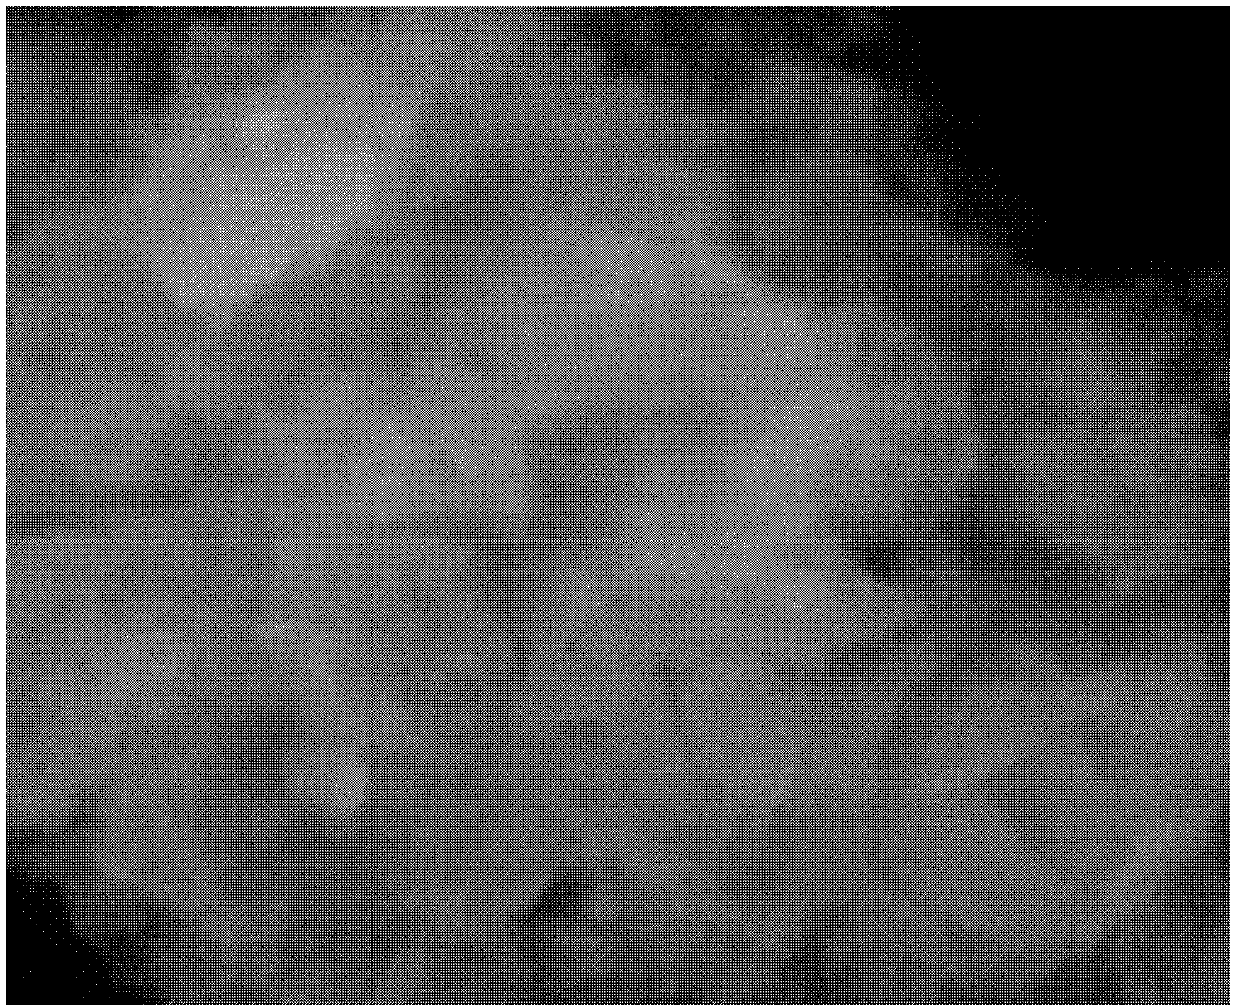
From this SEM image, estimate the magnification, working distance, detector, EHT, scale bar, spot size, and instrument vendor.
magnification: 20 K X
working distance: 7.6 mm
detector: LFD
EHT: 25 kV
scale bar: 3000 nm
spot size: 4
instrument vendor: FEI